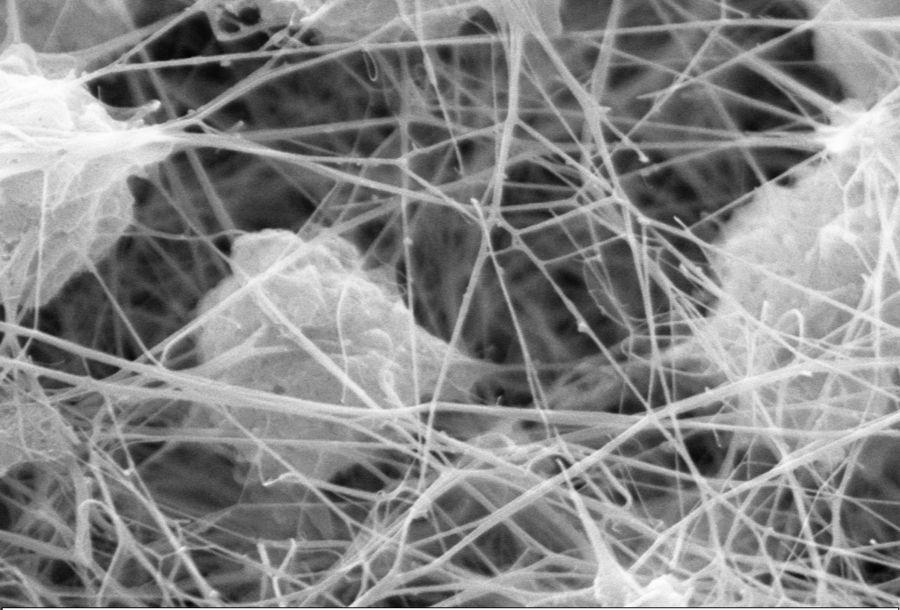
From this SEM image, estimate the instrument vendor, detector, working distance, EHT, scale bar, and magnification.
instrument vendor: Zeiss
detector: SE1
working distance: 7.98 mm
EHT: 15 kV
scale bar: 1000 nm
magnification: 50 K X